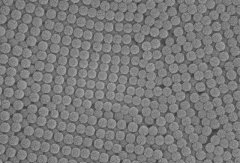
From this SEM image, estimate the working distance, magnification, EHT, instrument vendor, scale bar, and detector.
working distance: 5.8 mm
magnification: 50 K X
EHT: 10 kV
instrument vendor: Hitachi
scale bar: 1000 nm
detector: SE(UL)